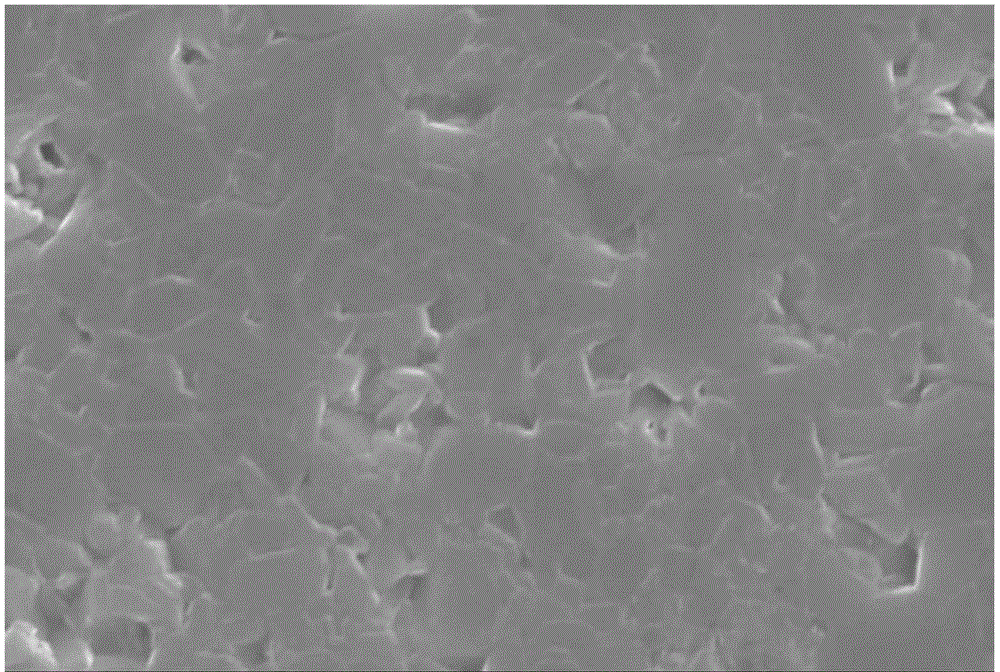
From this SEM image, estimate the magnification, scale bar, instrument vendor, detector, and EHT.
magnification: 2.2 K X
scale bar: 10000 nm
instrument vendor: JEOL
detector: SEI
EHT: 30 kV